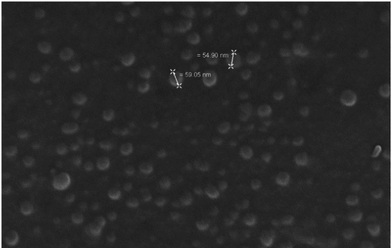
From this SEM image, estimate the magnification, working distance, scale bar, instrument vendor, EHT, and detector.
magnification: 202.01 K X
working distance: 2.5 mm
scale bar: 20 nm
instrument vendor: Zeiss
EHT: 5 kV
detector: InLens